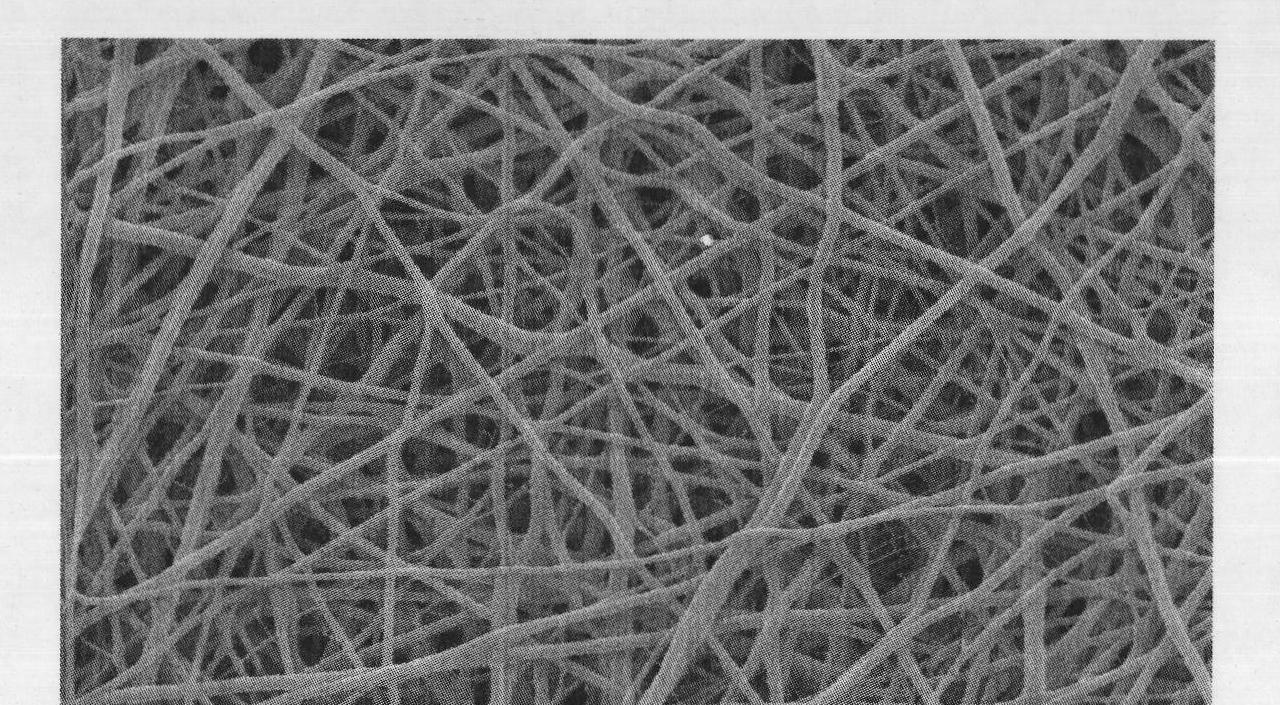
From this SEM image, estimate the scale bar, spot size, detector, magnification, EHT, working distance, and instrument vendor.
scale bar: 5000 nm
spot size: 3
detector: SE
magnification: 5 K X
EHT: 10 kV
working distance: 4.9 mm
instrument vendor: FEI